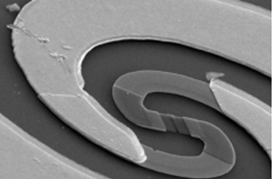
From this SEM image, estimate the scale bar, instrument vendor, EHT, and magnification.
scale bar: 5000 nm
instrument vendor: JEOL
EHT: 20 kV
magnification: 4.5 K X